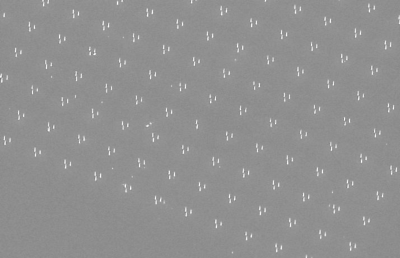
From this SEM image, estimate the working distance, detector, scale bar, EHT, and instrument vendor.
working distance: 10 mm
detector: SE1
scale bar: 20000 nm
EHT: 15 kV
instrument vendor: Zeiss